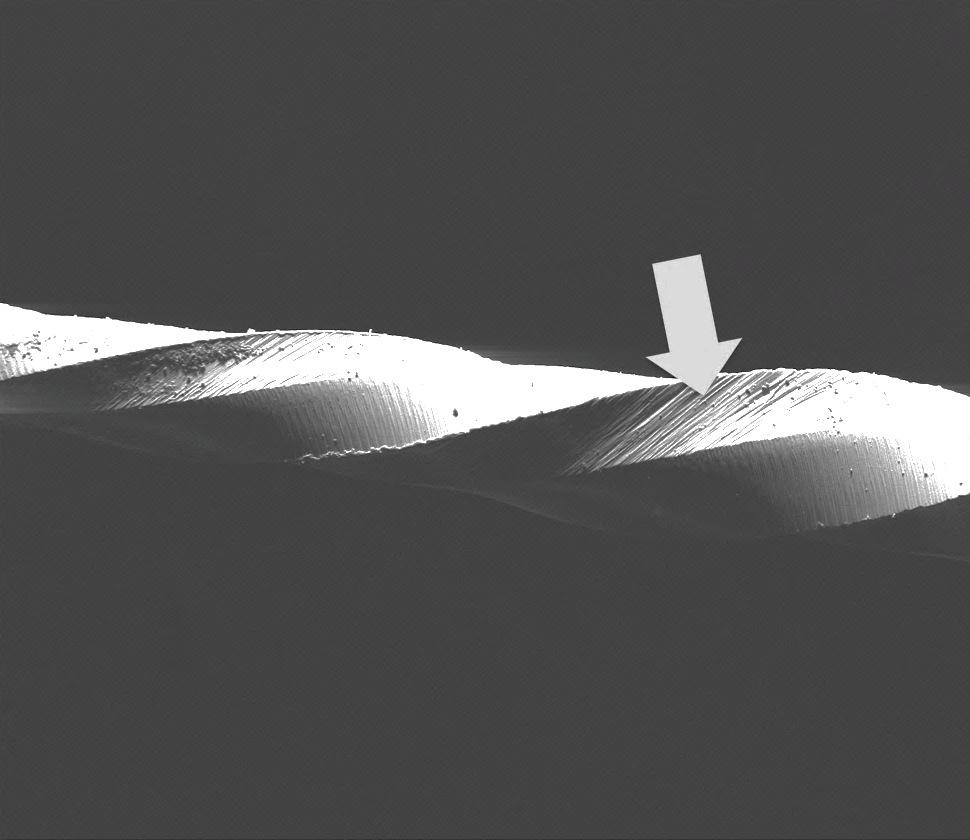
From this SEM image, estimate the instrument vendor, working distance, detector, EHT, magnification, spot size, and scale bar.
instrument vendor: FEI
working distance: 11.9 mm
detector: ETD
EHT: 30 kV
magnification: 0.15 K X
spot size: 6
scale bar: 1000000 nm